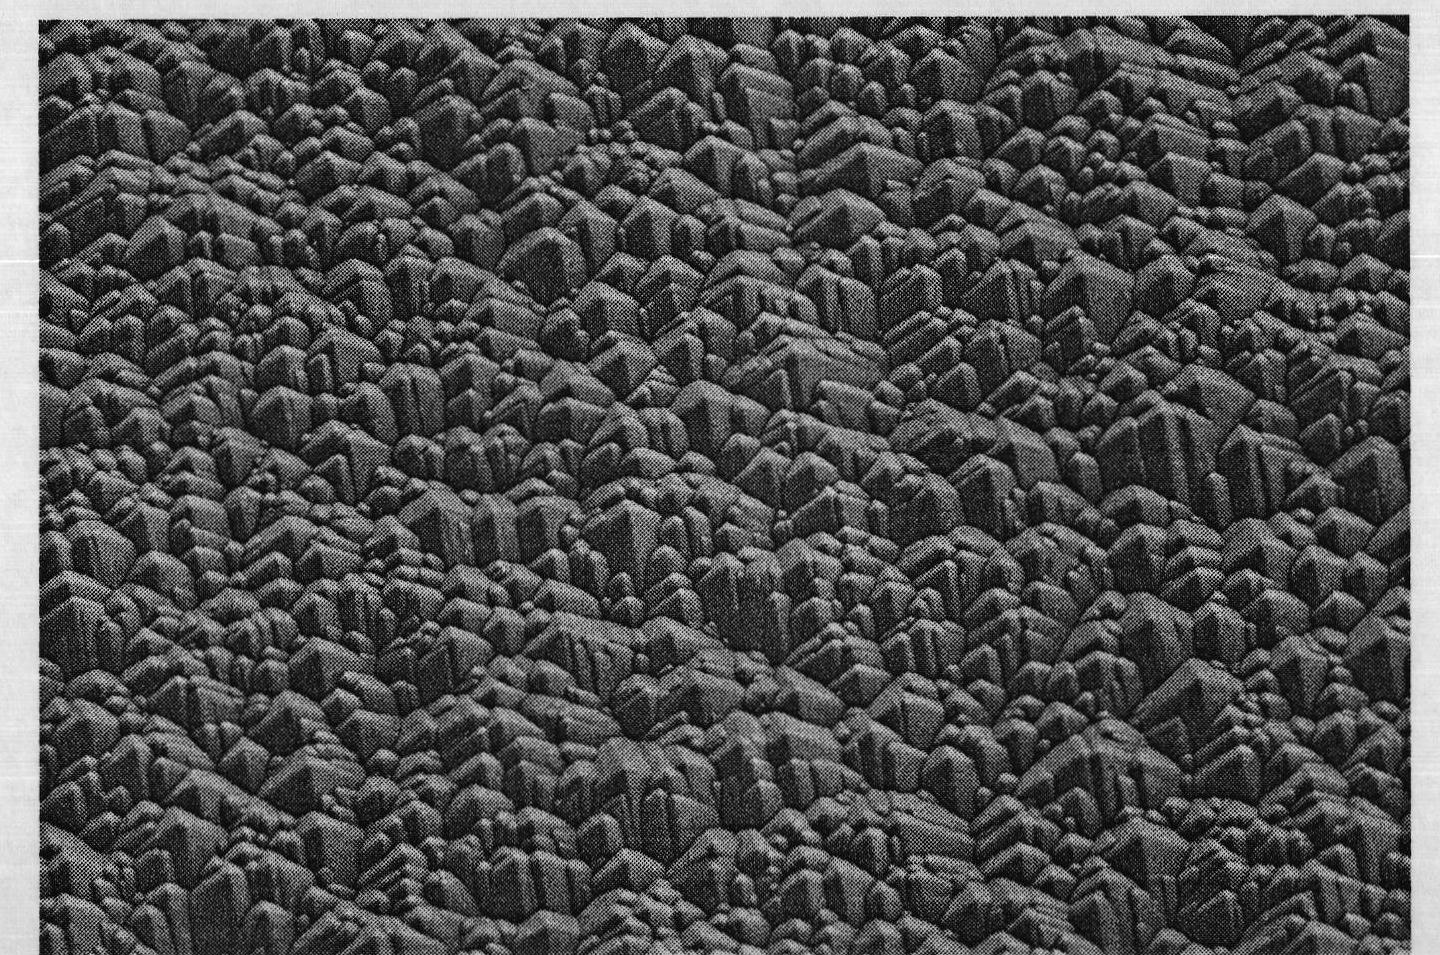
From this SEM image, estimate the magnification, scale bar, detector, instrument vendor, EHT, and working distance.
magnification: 2 K X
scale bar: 20000 nm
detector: SE(M)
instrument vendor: Hitachi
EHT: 5 kV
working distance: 9 mm